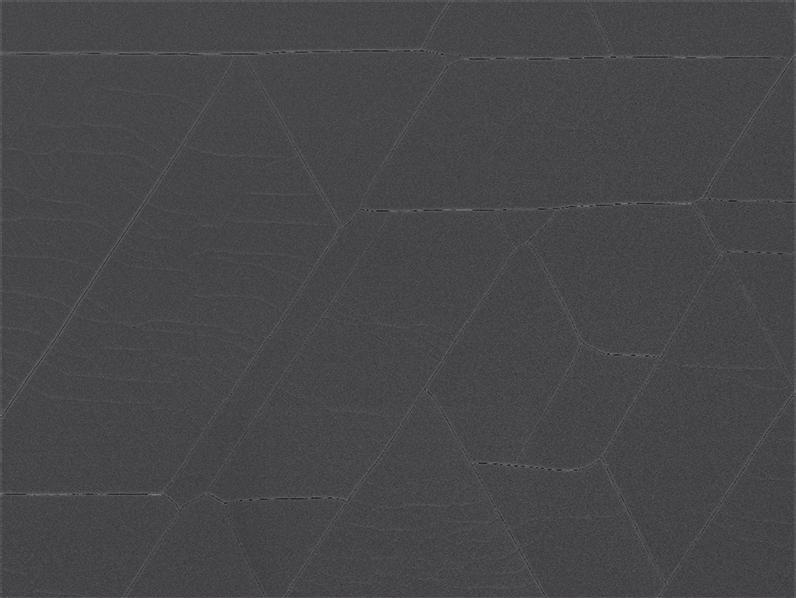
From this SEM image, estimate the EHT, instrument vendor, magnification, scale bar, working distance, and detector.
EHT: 15 kV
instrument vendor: JEOL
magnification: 0.33 K X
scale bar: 10000 nm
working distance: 14.7 mm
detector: SEI